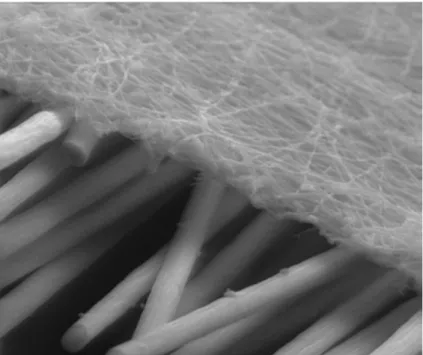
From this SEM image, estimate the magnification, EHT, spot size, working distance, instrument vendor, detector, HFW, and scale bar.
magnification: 1.3 K X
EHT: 25 kV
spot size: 3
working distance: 8.4 mm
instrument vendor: FEI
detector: LFD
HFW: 100 µm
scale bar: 50000 nm